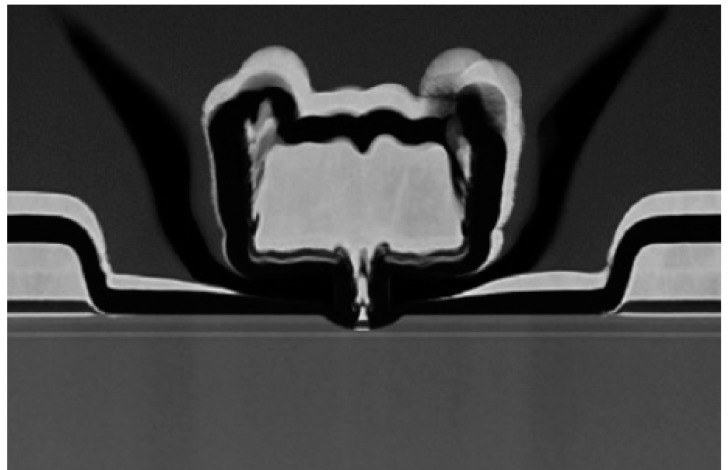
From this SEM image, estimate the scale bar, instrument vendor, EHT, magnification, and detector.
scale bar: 300 nm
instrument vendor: Hitachi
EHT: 200 kV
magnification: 100 K X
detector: ZC